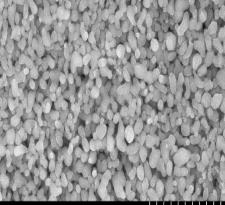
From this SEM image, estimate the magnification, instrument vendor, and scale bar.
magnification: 20 K X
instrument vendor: Hitachi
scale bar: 2000 nm